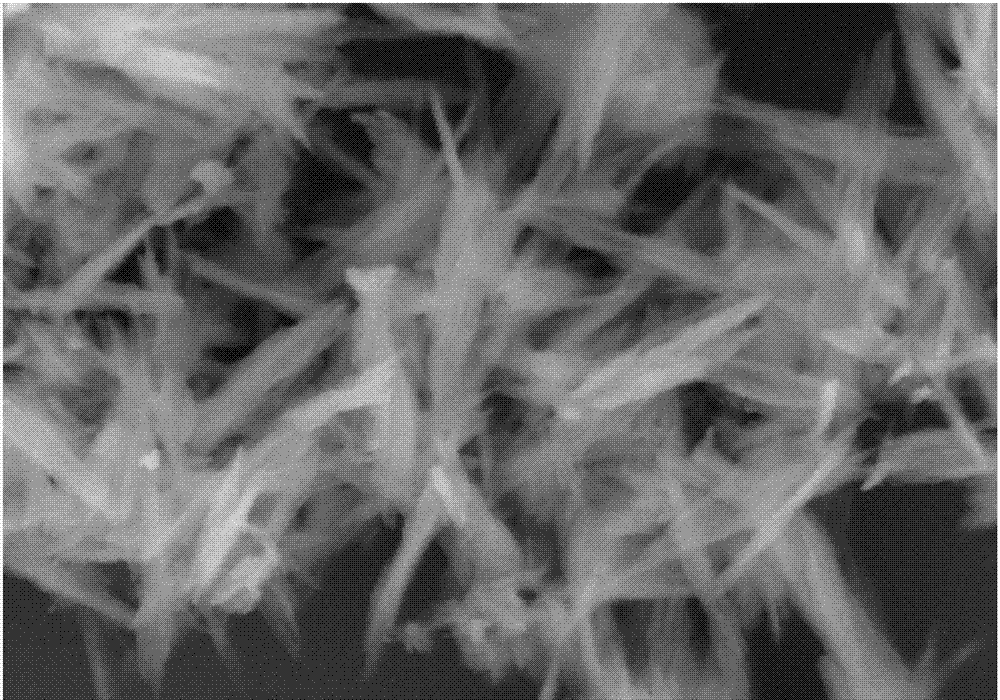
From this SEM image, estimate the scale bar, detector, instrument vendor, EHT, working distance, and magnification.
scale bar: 500 nm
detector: SE(U,LA0)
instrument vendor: Hitachi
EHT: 5 kV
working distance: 3.3 mm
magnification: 100 K X